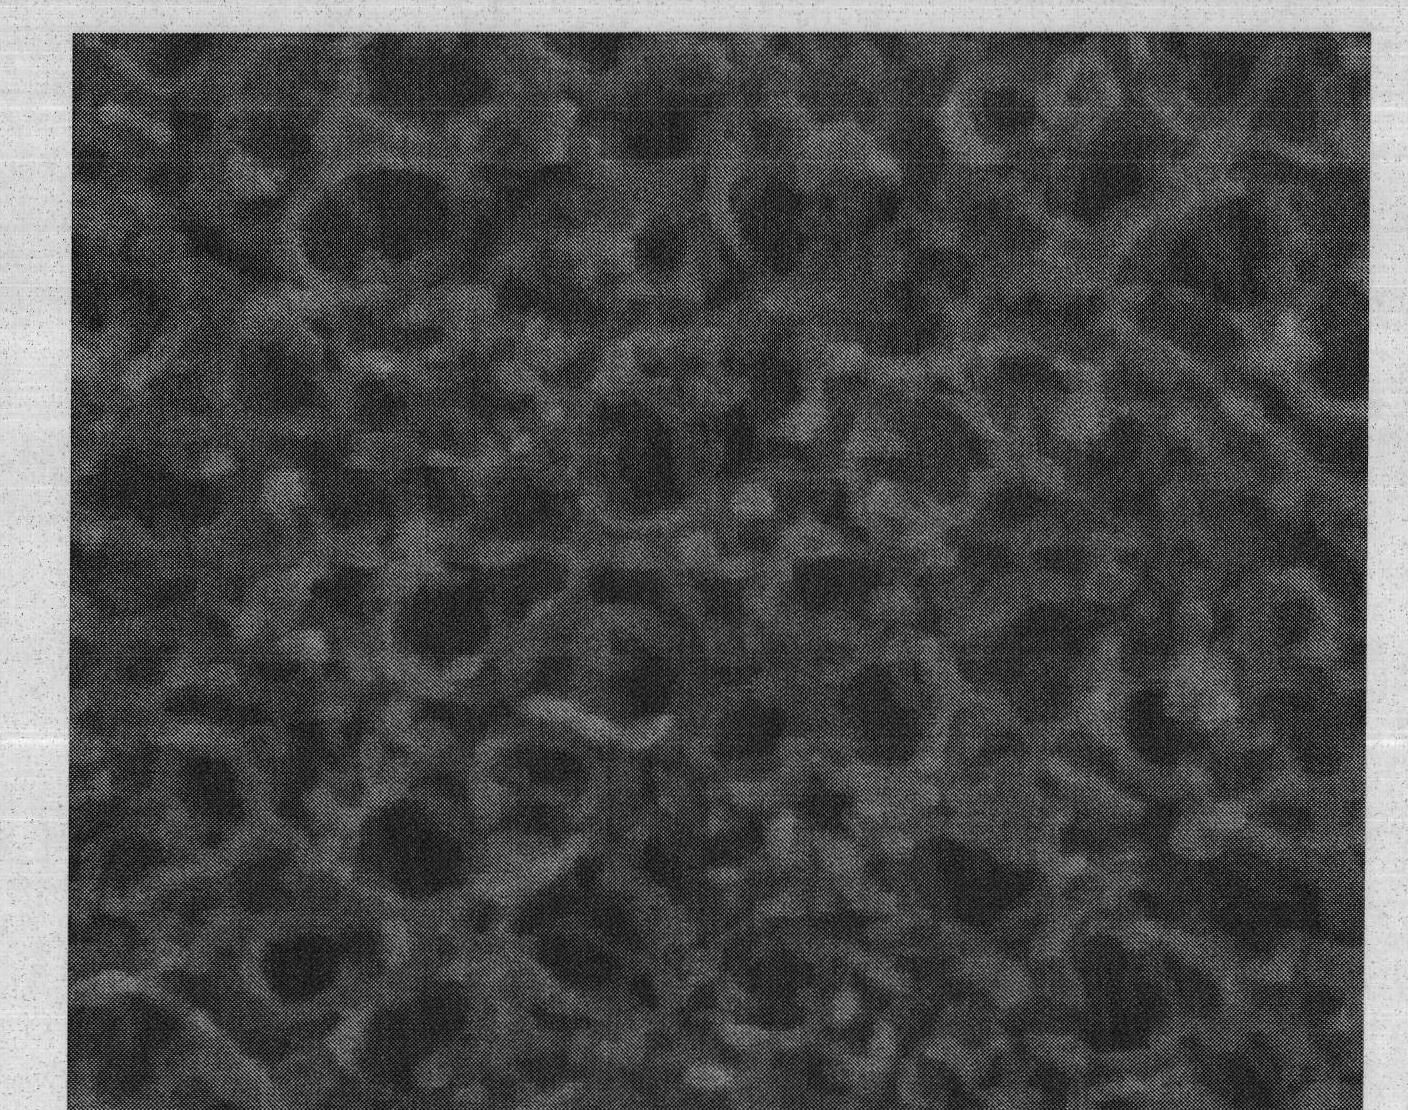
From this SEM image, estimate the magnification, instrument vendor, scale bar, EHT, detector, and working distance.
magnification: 200 K X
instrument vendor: FEI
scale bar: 300 nm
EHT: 10 kV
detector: TLD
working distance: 5.7 mm